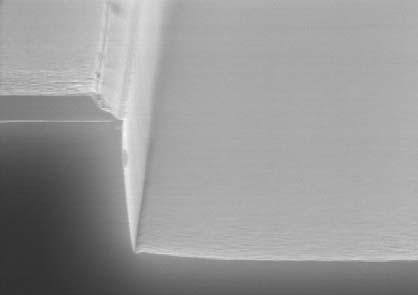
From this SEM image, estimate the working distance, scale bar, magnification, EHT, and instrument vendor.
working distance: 11.4 mm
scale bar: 1000 nm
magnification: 30 K X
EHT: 15 kV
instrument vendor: Hitachi